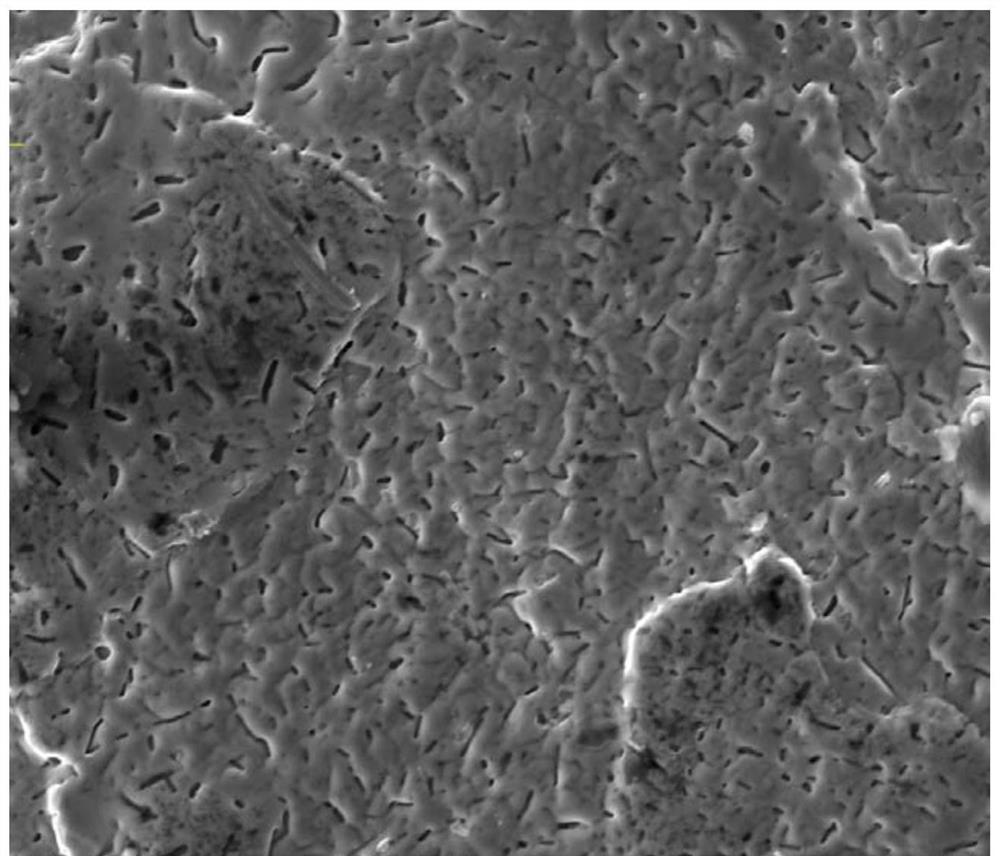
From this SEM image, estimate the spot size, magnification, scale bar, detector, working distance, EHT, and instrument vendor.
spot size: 4.5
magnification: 2 K X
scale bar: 50000 nm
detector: SE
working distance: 19.6 mm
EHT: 30 kV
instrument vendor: FEI